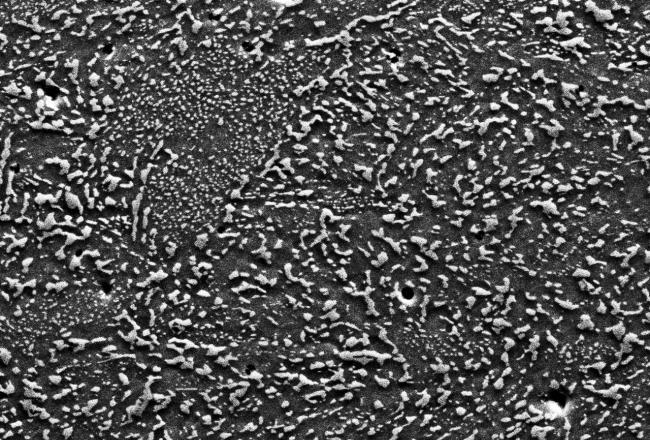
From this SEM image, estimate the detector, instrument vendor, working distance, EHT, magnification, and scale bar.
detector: SE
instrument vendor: Hitachi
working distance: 22.4 mm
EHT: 20 kV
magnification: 2.5 K X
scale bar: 20000 nm